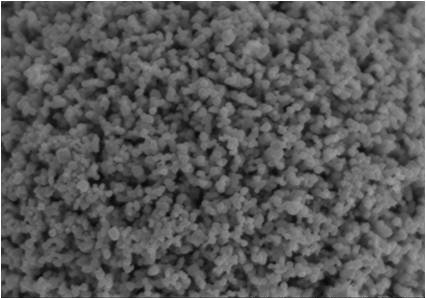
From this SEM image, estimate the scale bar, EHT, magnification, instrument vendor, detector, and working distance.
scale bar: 10000 nm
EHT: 20 kV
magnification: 5 K X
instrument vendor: Hitachi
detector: SE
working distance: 6.3 mm